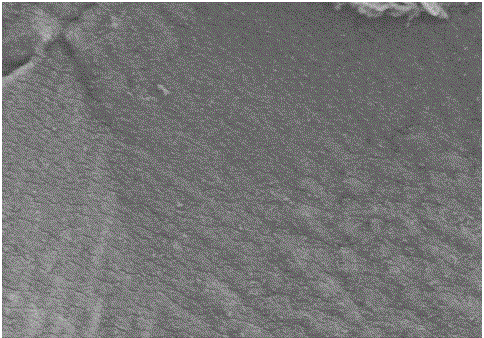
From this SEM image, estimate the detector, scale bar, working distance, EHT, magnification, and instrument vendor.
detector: SE(M)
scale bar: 1000 nm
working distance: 9.7 mm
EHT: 5 kV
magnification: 30 K X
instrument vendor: Hitachi